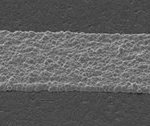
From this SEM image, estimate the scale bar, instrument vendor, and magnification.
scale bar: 100000 nm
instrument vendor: JEOL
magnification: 0.12 K X